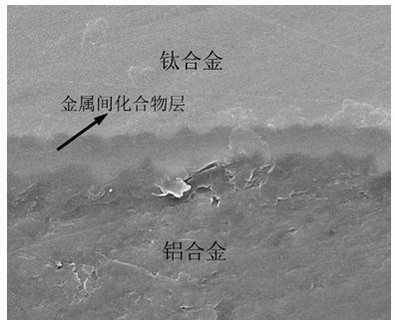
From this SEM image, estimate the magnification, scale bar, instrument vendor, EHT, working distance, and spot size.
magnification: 10 K X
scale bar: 10000 nm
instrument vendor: FEI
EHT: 20 kV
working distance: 10.1 mm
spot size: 3.5